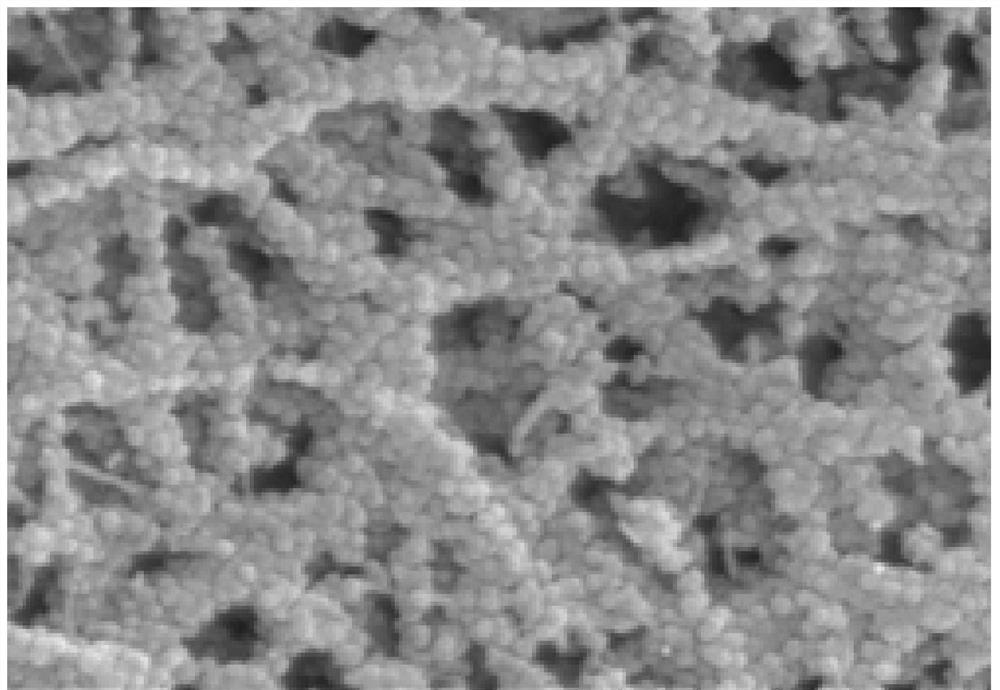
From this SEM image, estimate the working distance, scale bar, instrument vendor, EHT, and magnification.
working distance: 13.5 mm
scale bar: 500 nm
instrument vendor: Hitachi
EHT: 5 kV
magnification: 100 K X